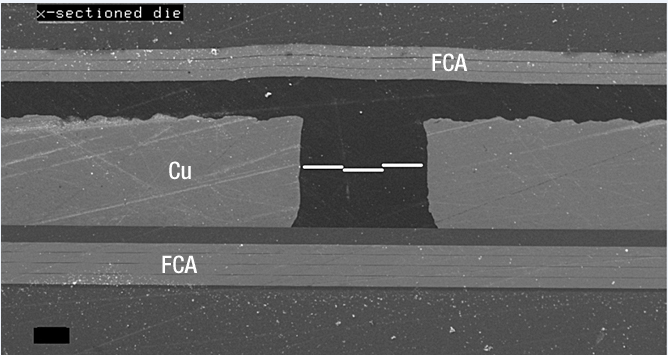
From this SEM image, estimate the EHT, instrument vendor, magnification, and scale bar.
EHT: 5 kV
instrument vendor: other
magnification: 0.68 K X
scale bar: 10000 nm